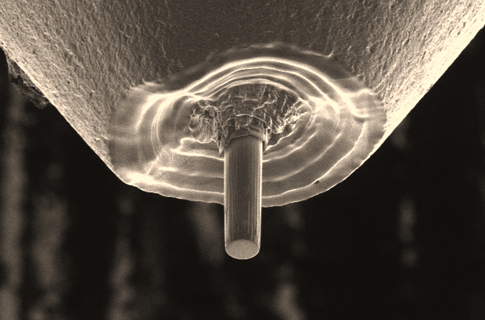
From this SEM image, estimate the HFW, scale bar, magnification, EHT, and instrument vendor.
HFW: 59.2 µm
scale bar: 20000 nm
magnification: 3.5 K X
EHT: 30 kV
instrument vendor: FEI